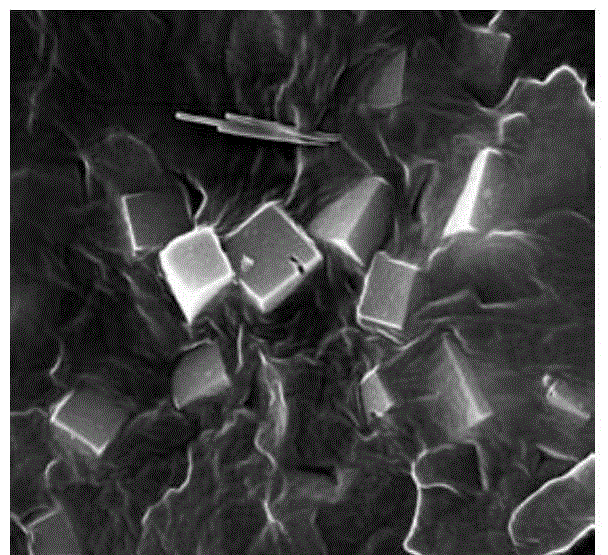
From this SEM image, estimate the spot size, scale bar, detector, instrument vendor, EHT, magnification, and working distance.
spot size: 3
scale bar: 5000 nm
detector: ETD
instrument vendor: FEI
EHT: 20 kV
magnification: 20 K X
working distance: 9.5 mm